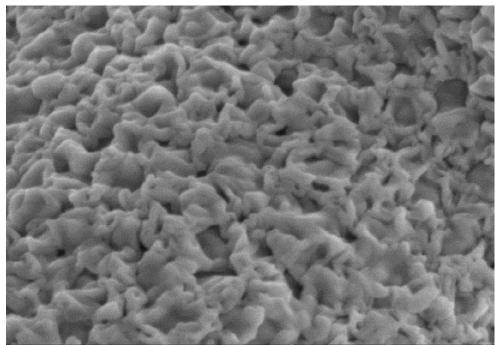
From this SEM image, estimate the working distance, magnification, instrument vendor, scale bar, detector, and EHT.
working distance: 11.3 mm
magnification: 50 K X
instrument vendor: Hitachi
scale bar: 1000 nm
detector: SE(UL)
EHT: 5 kV